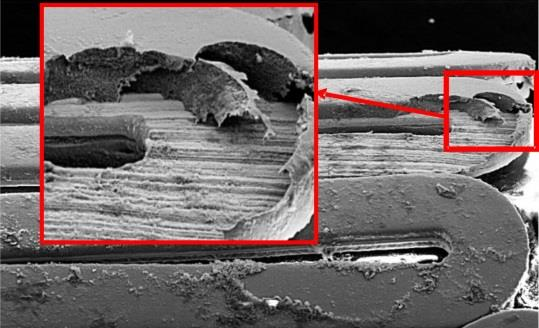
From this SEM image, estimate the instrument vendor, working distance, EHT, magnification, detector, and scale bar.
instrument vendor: Zeiss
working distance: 16 mm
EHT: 20 kV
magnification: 0.4 K X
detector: SE2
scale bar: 100000 nm